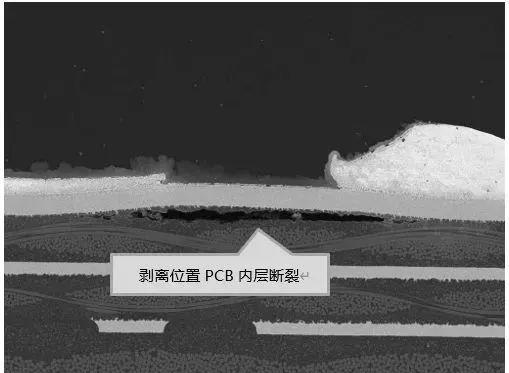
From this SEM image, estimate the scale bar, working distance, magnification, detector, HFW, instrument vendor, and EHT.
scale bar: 200000 nm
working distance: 12.2 mm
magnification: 0.235 K X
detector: BSE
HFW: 1180 µm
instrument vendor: Tescan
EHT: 20 kV